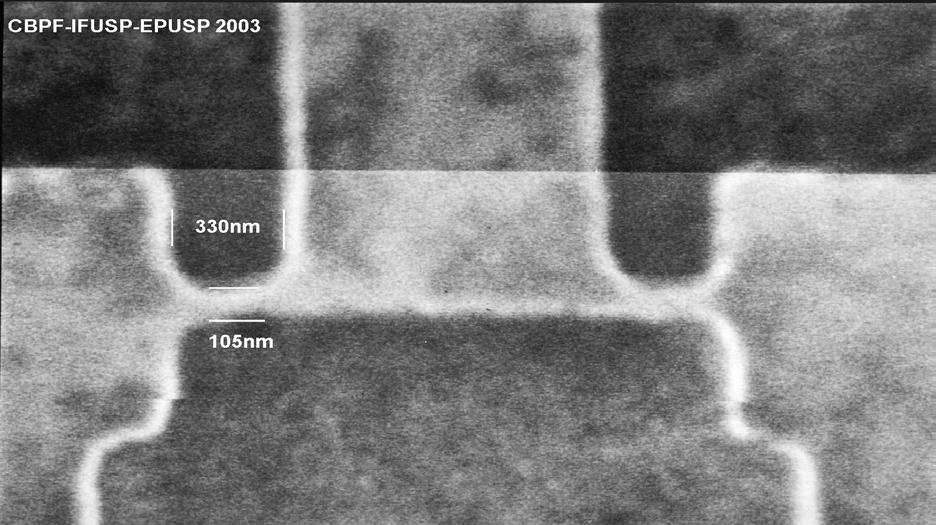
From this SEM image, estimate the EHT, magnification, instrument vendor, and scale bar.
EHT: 29.9 kV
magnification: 40 K X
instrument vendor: JEOL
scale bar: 1000 nm